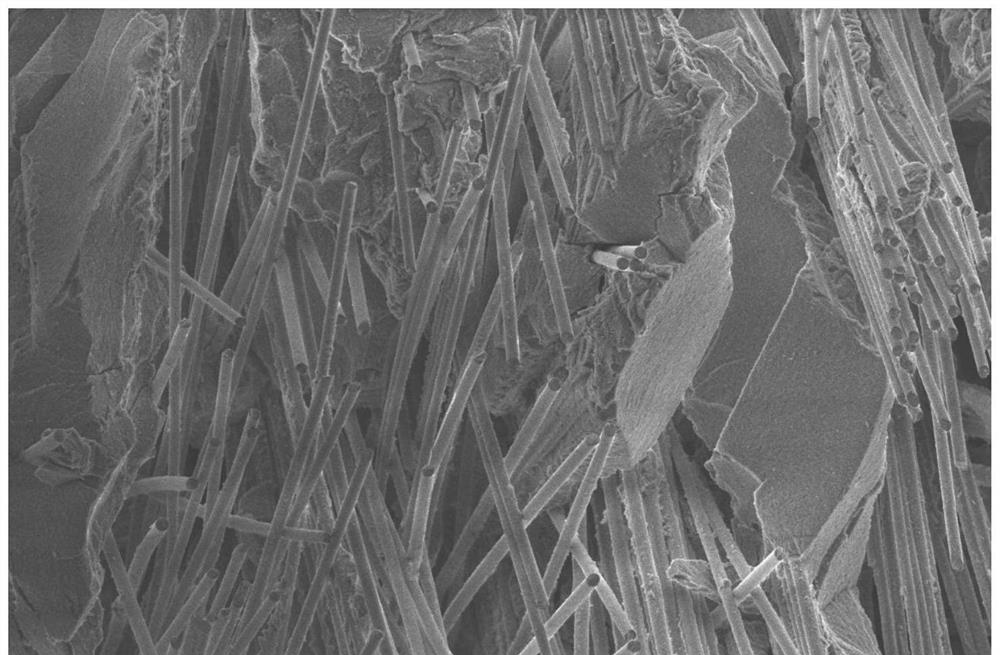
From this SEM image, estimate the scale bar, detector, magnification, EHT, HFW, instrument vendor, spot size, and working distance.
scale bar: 100000 nm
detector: ETD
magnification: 0.65 K X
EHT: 3 kV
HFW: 638 µm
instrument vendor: Thermo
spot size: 4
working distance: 9.9 mm